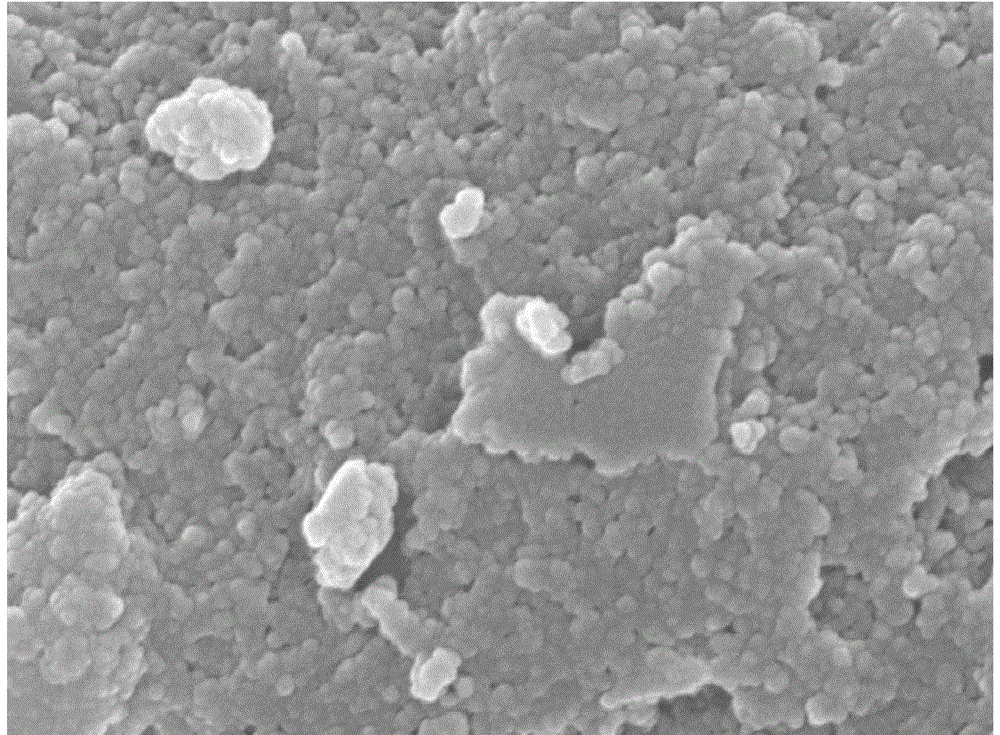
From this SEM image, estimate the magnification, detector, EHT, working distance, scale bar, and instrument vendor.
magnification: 50 K X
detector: SEM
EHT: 15 kV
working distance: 8.1 mm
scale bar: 100 nm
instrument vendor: JEOL